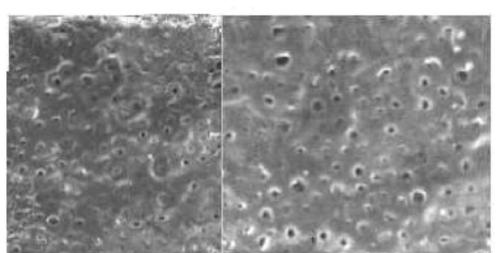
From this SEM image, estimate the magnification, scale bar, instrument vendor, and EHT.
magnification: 2 K X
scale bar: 10000 nm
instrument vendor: JEOL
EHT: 8 kV